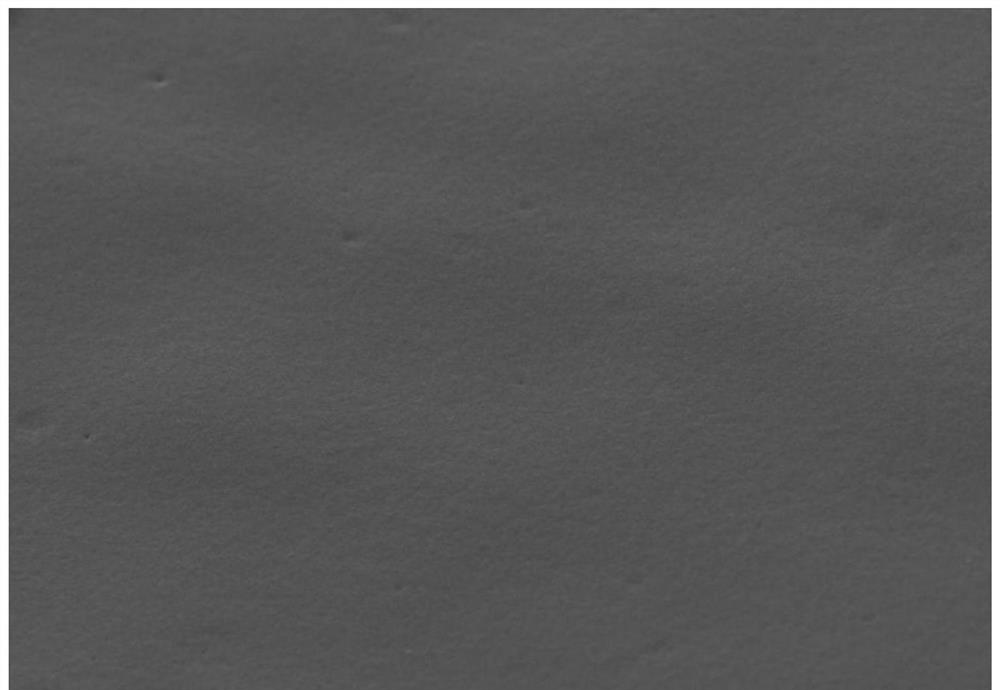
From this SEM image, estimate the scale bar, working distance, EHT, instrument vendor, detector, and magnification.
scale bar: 20000 nm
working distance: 8.7 mm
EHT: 15 kV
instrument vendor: Hitachi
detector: SE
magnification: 2 K X